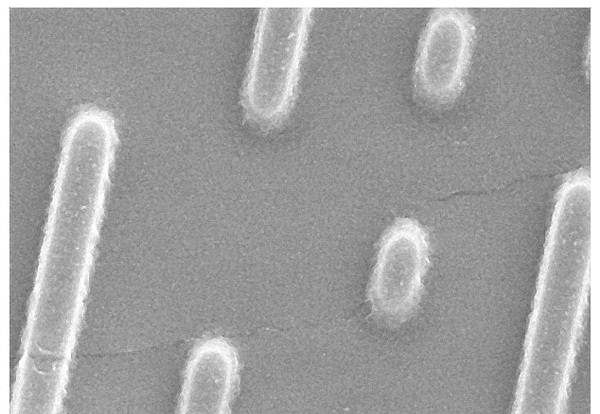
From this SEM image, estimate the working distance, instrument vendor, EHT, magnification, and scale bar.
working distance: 6 mm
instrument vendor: other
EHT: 5 kV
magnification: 20 K X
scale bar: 2000 nm